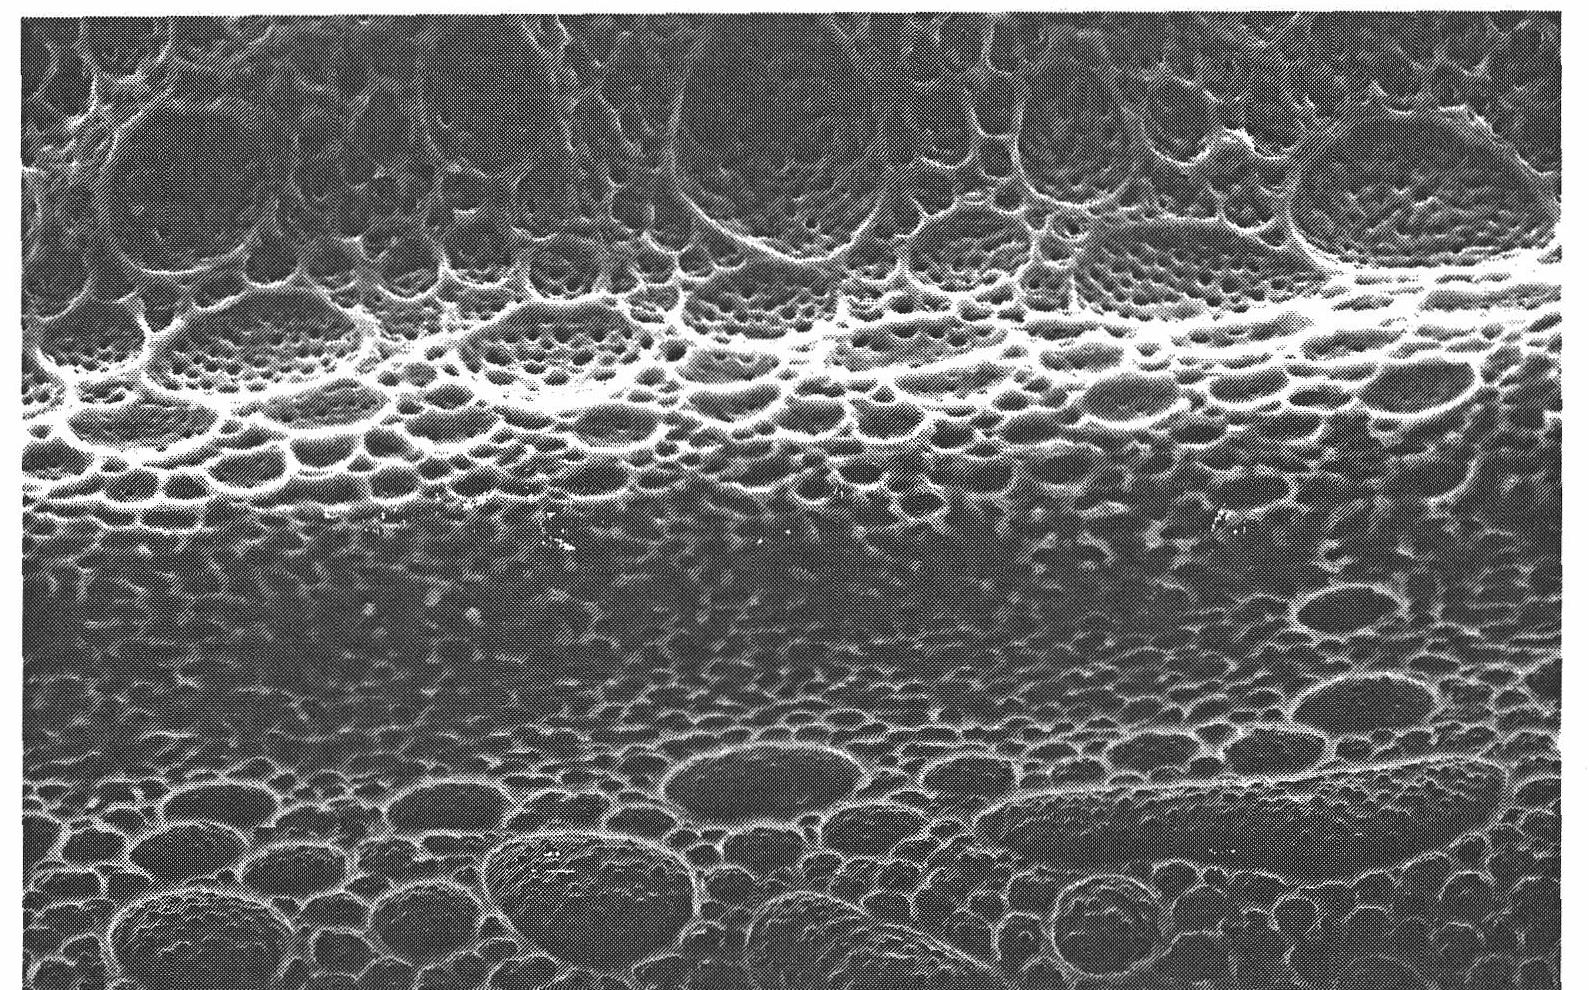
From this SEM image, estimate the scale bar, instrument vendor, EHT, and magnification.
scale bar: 50000 nm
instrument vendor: JEOL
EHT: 15 kV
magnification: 0.5 K X